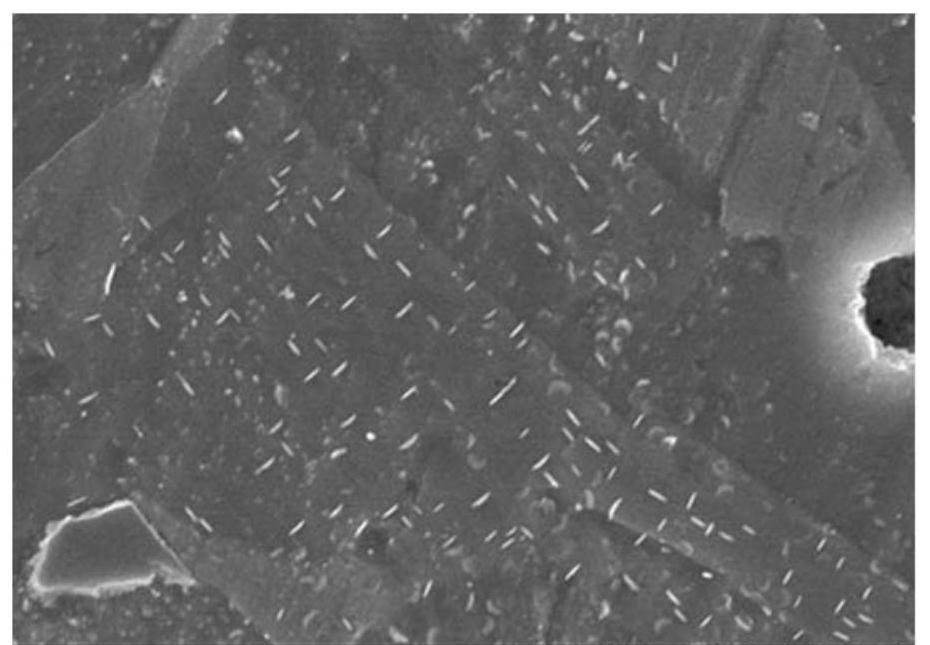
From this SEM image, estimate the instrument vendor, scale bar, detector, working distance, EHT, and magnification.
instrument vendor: Hitachi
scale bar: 5000 nm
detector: SE(M)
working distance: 8.5 mm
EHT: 20 kV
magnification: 10 K X